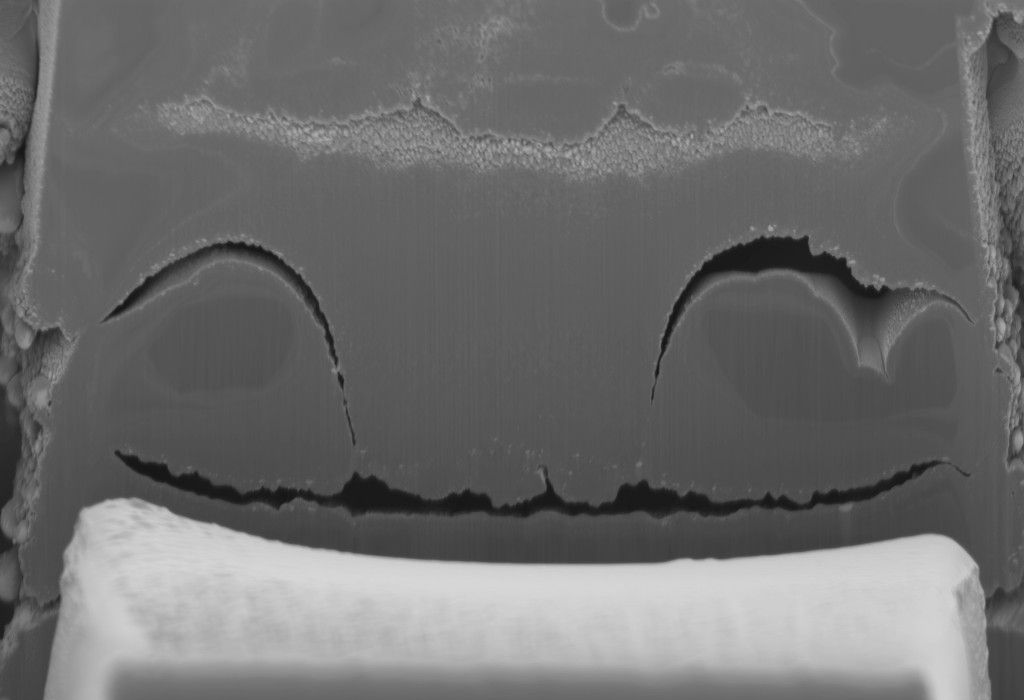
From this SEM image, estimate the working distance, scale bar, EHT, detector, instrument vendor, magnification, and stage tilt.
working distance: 5 mm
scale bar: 2000 nm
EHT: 2 kV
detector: InLens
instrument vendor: Zeiss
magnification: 4.52 K X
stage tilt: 0°